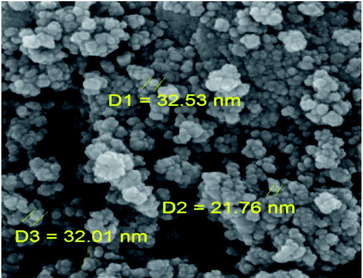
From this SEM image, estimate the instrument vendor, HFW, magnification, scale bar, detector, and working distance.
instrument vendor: Tescan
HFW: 1.04 µm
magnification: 200 K X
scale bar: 200 nm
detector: InBeam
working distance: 4.95 mm